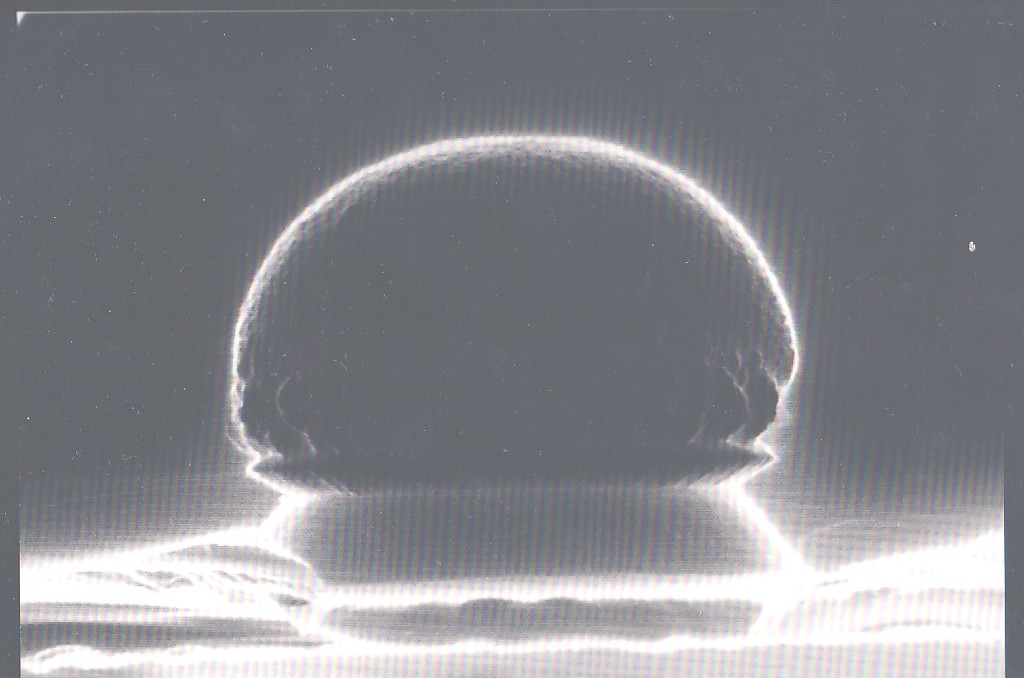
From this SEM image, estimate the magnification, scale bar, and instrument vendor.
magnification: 50 K X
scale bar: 1000 nm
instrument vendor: FEI